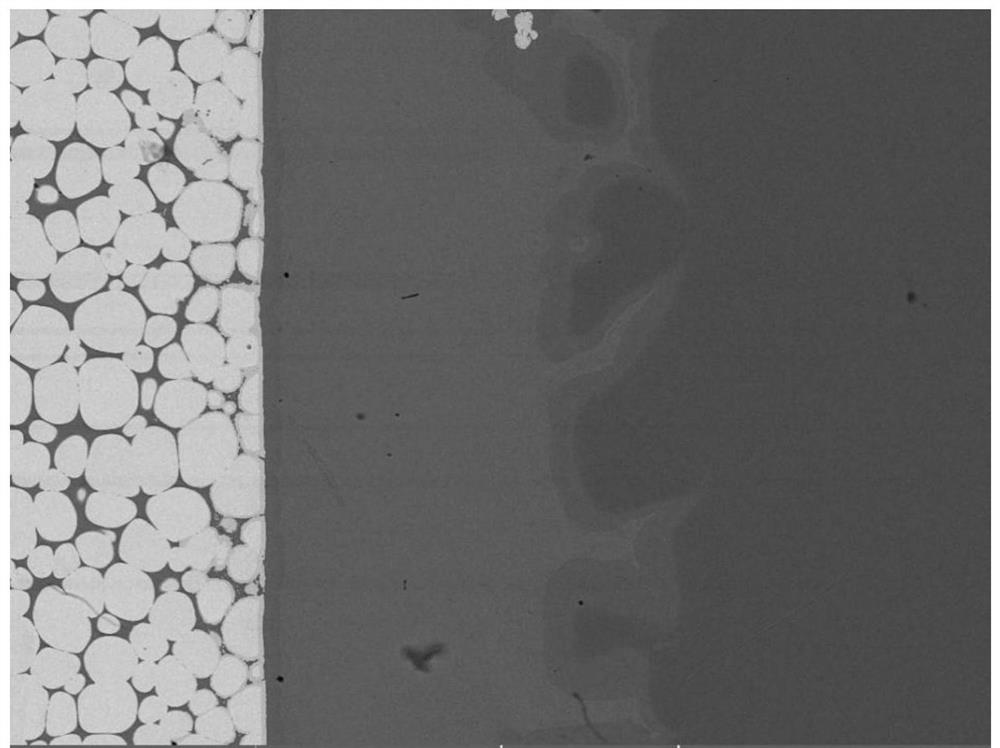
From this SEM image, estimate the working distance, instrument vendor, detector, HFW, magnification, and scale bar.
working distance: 12.23 mm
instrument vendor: Tescan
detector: BSE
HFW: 554 µm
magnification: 0.5 K X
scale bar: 100000 nm